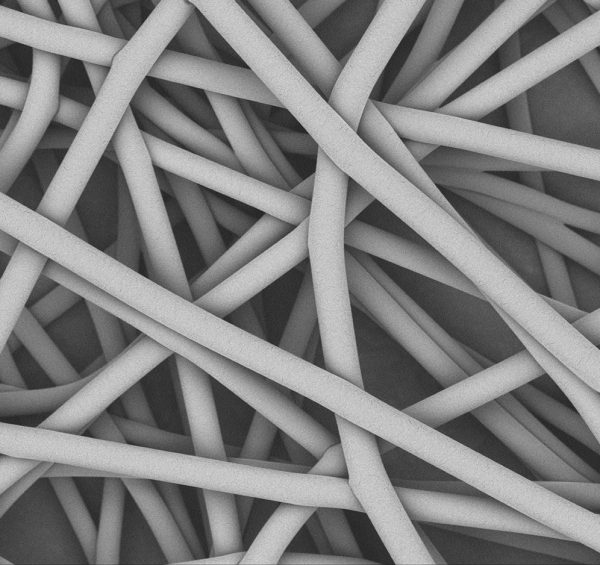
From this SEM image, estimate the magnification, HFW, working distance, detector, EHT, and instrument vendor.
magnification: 5 K X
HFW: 120 µm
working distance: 6.696 mm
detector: BSD Full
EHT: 10 kV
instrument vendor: Phenom